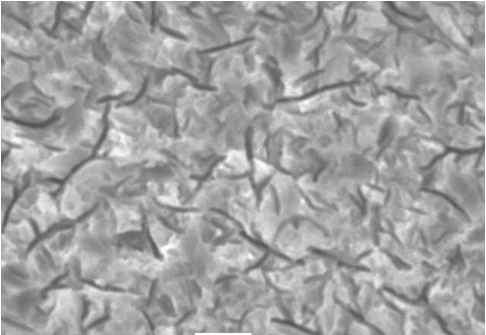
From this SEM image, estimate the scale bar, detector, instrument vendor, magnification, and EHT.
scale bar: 5000 nm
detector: SEI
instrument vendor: JEOL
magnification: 3 K X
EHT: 20 kV